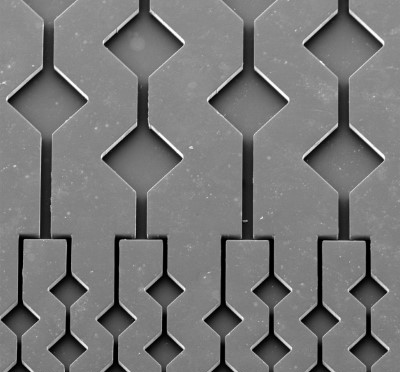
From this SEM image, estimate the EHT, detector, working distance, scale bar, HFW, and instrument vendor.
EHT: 10 kV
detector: SE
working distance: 16.43 mm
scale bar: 200000 nm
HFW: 1540 µm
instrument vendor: Tescan